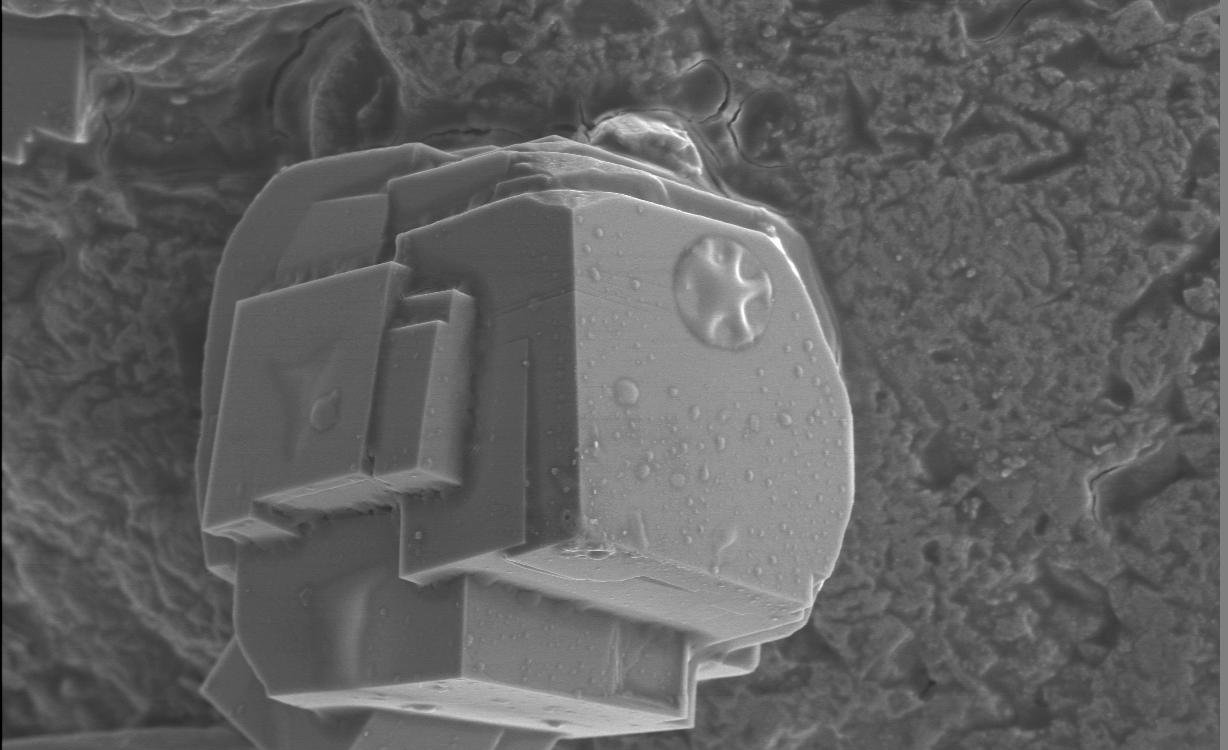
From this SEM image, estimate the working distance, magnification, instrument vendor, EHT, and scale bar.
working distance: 11 mm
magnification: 2.5 K X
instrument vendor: JEOL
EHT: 7 kV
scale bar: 10000 nm